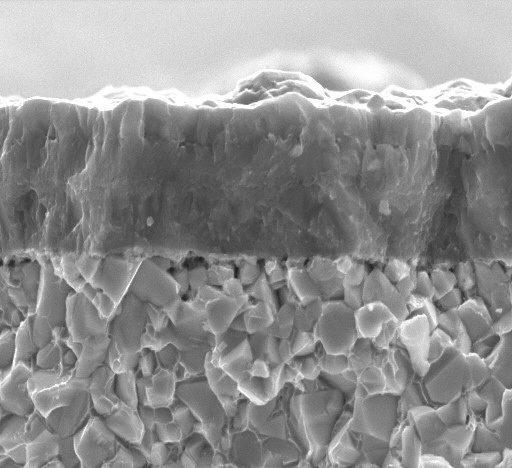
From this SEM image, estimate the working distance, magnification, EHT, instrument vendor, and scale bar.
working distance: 8 mm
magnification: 5 K X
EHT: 20 kV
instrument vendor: JEOL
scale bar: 5000 nm